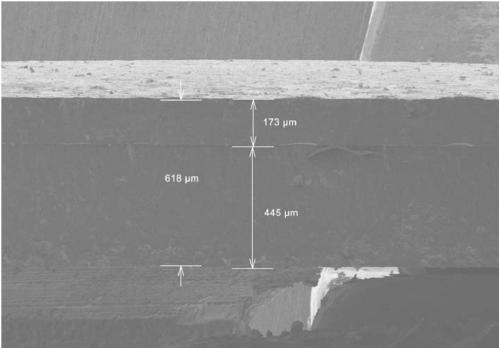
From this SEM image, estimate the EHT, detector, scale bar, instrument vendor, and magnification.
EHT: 4 kV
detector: SE(M)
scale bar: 500000 nm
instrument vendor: Hitachi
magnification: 0.07 K X